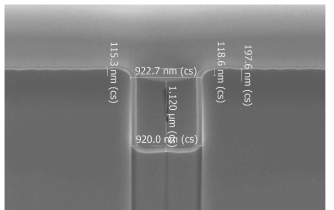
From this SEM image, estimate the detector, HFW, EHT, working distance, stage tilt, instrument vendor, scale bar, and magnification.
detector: TLD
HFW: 4.14 µm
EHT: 5 kV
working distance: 4 mm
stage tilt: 55°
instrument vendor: FEI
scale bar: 1000 nm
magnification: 50 K X